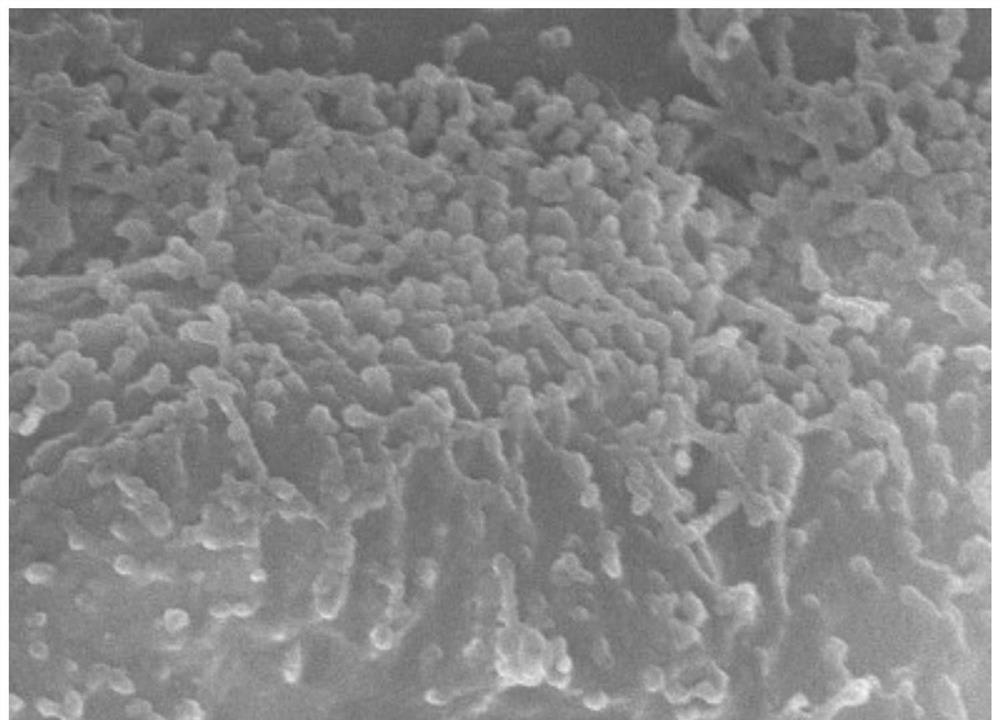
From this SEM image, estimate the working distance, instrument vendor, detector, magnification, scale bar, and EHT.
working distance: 8 mm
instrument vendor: JEOL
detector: SEI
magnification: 20 K X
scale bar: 1000 nm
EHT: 15 kV